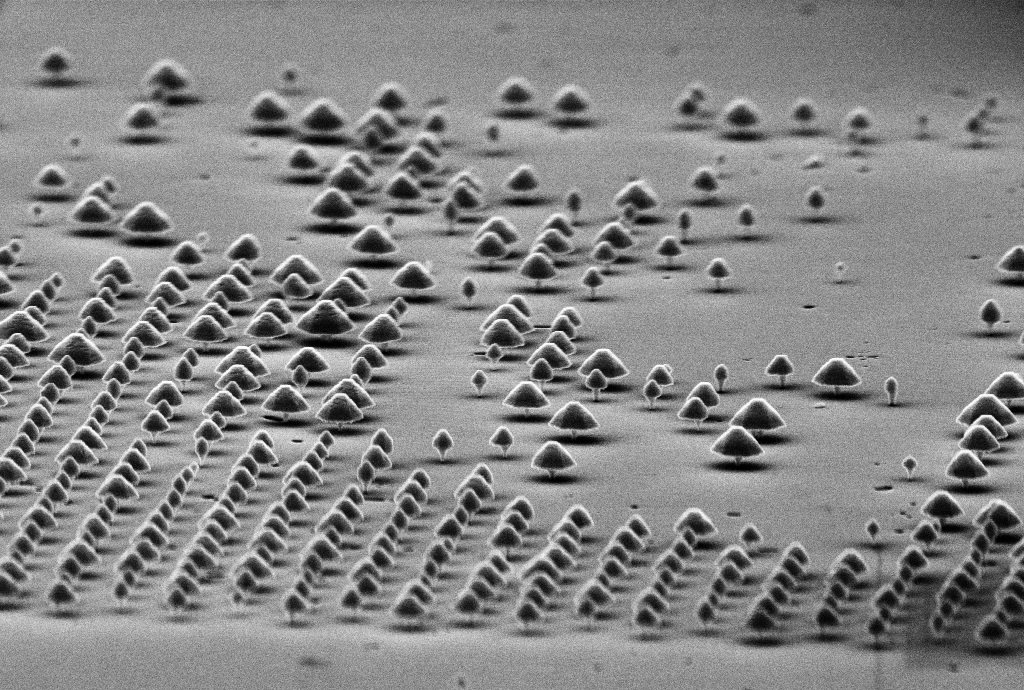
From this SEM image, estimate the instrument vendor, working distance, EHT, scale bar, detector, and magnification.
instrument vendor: Zeiss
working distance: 13.4 mm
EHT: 10 kV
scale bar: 1000 nm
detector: SE2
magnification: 14.16 K X